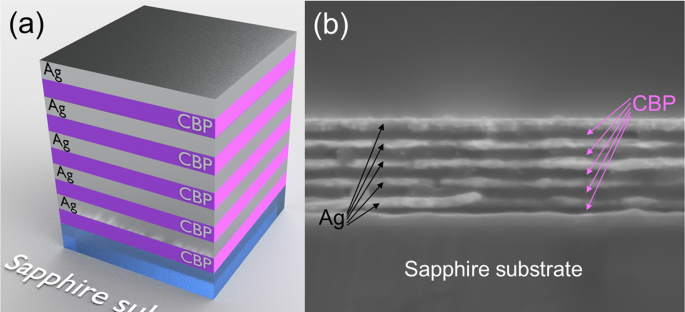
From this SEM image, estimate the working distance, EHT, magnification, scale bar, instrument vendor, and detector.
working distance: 5.6 mm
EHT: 3.5 kV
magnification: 158.17 K X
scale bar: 200 nm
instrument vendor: Zeiss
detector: InLens